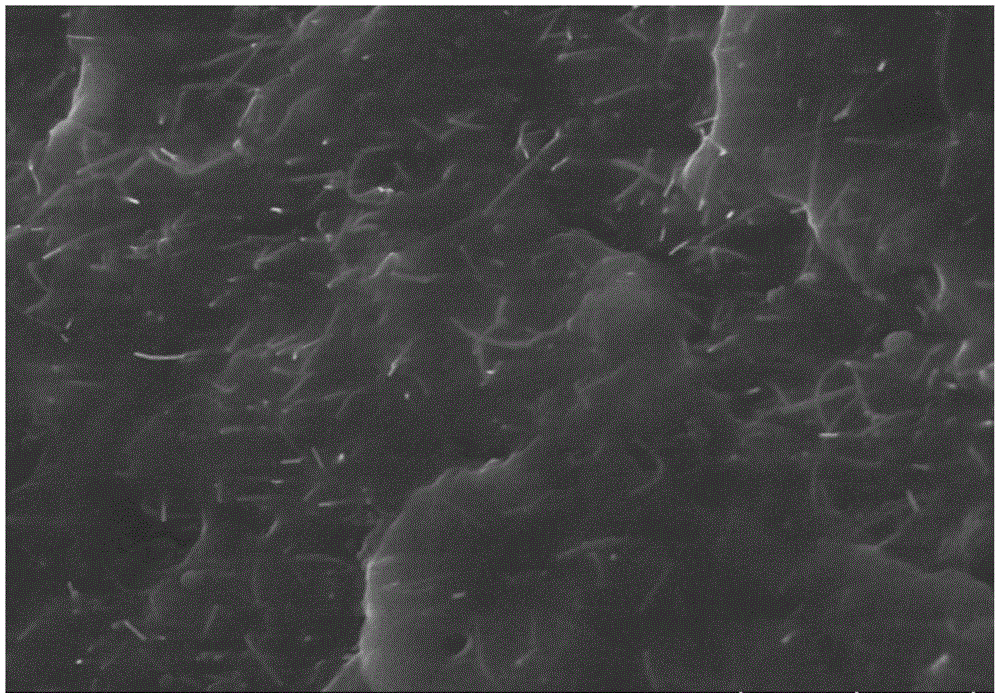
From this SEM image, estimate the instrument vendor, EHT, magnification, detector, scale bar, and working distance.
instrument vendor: Hitachi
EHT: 5 kV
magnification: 30 K X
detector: SE(U)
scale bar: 1000 nm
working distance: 8.5 mm